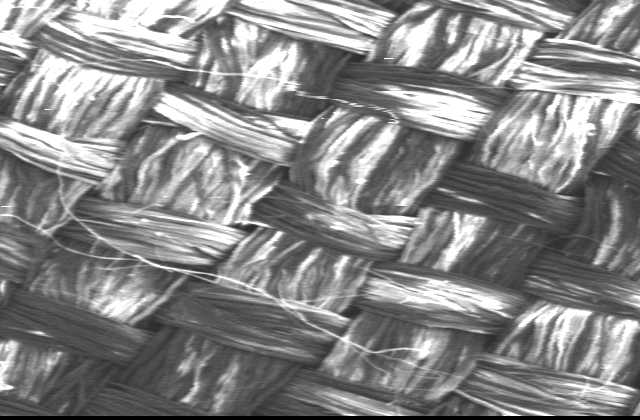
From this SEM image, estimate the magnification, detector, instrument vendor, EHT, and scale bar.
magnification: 0.04 K X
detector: SEI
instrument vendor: JEOL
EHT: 13 kV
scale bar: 500000 nm